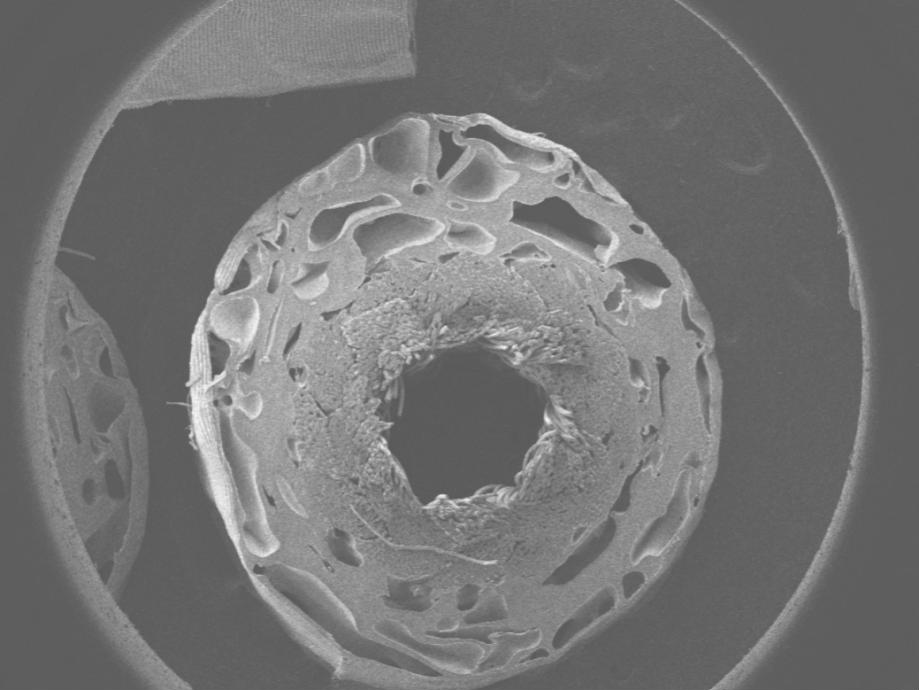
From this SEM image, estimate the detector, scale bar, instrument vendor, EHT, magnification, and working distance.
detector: LEI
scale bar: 1000000 nm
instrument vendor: JEOL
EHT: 10 kV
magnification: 0.025 K X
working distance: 8 mm